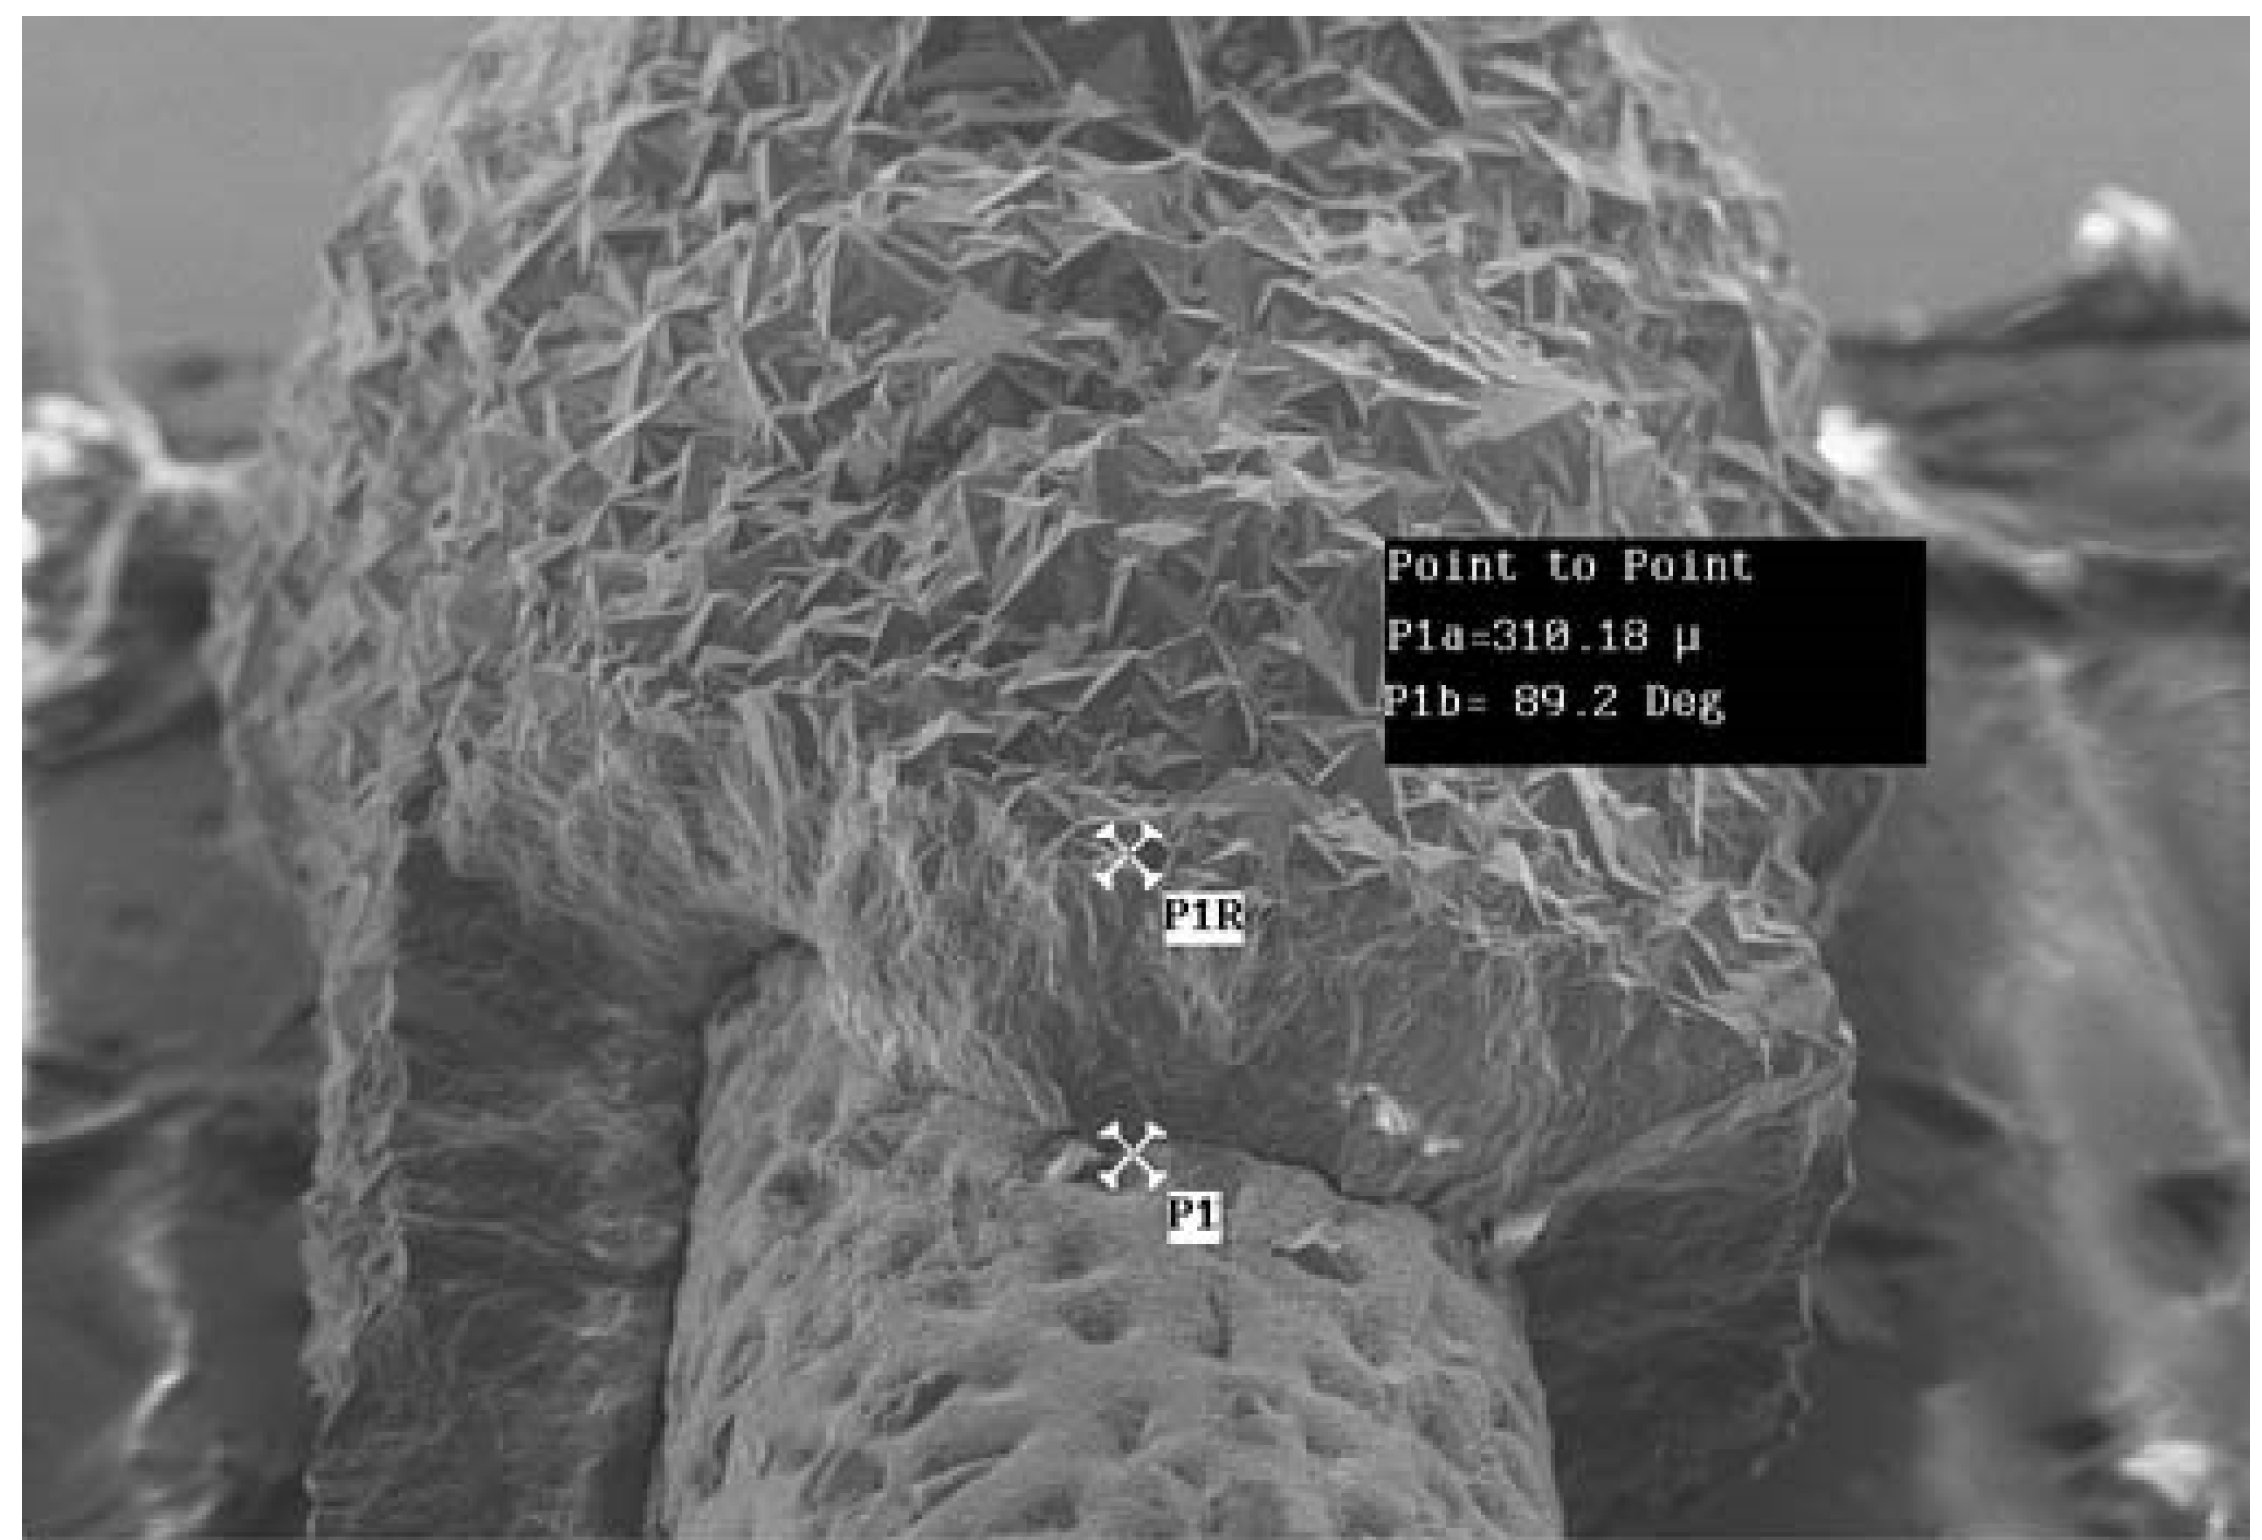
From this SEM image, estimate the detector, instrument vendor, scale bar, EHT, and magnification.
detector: SE1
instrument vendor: Zeiss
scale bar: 40000 nm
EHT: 20 kV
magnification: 0.075 K X